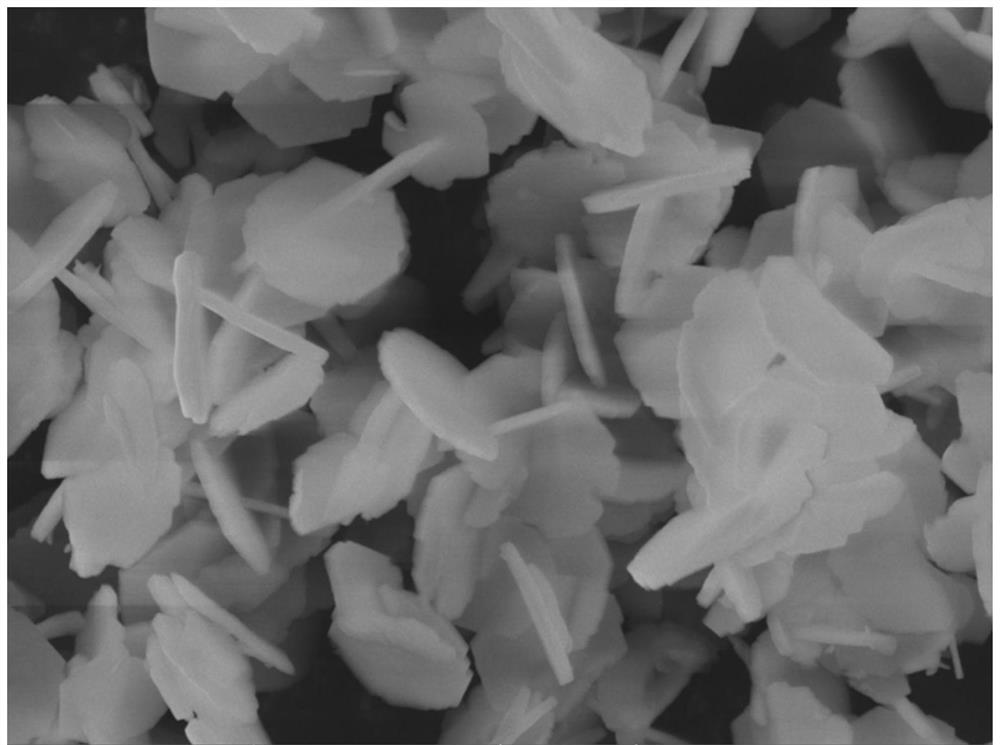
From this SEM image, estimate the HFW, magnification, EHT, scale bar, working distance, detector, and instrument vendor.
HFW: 14.6 µm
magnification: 19 K X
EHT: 10 kV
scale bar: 2000 nm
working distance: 7.51 mm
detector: In-Beam SE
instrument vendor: Tescan